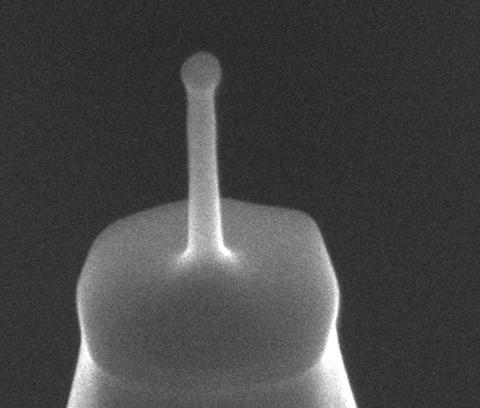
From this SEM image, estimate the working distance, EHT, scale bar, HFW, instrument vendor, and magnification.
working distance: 4 mm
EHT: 5 kV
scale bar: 200 nm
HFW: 0.635 µm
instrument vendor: FEI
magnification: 200 K X